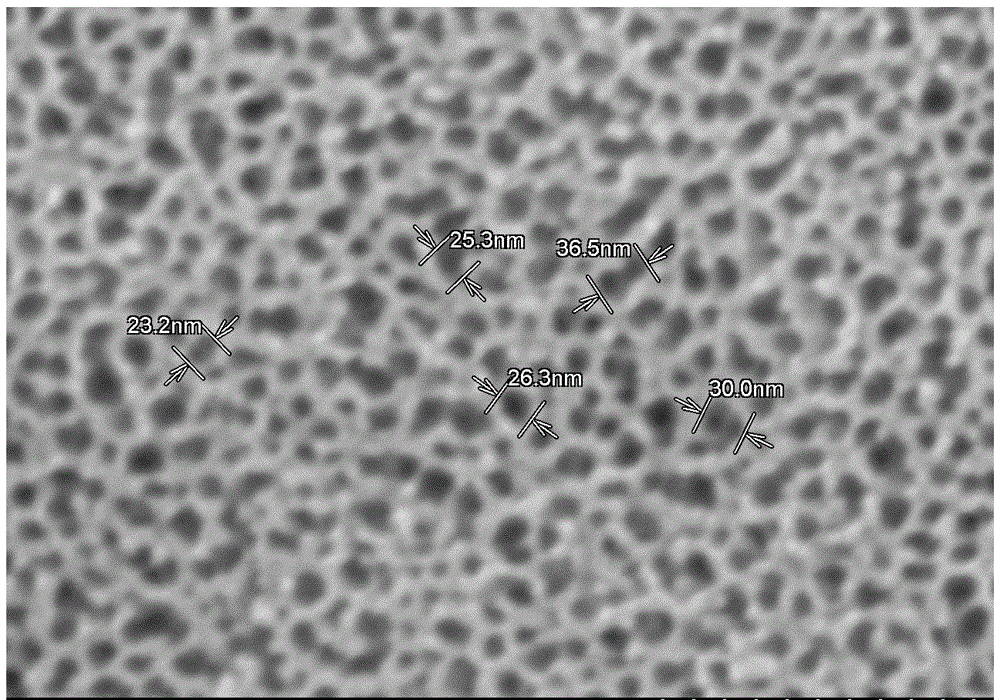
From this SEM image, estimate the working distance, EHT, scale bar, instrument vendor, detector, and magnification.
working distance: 4 mm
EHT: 5 kV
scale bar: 200 nm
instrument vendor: Hitachi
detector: SE(M)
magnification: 200 K X